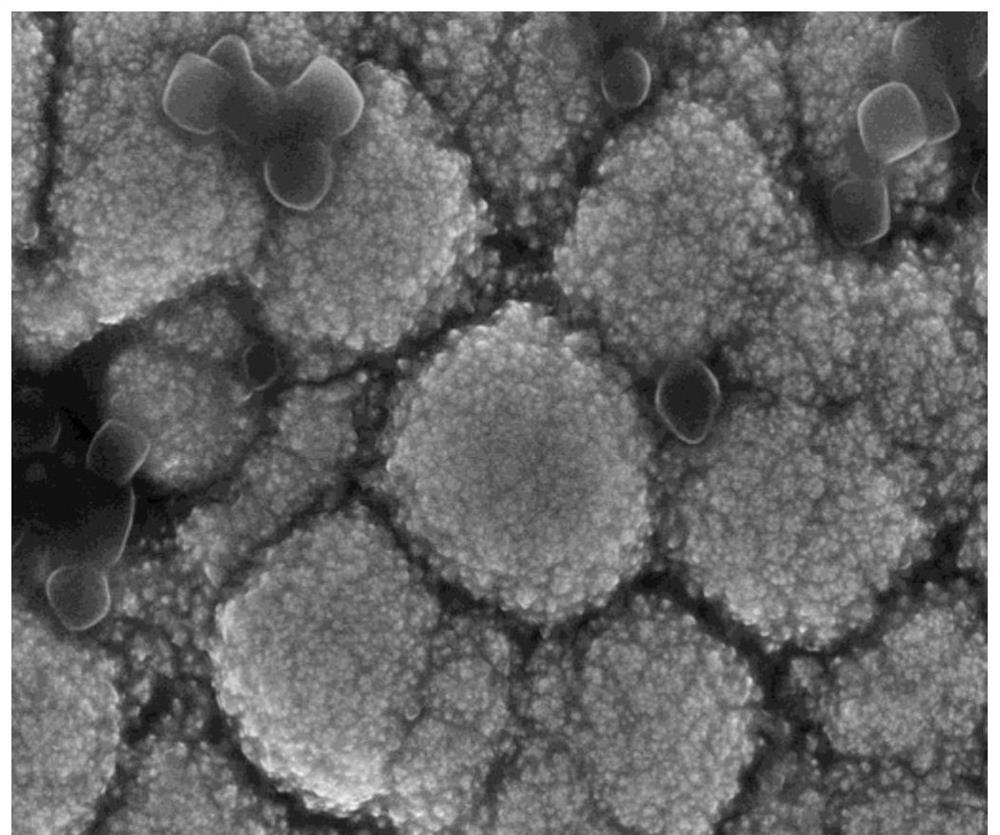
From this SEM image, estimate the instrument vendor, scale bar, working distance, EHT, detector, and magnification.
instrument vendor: Hitachi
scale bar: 2000 nm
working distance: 8.1 mm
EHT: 15 kV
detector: SE(U)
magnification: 20 K X